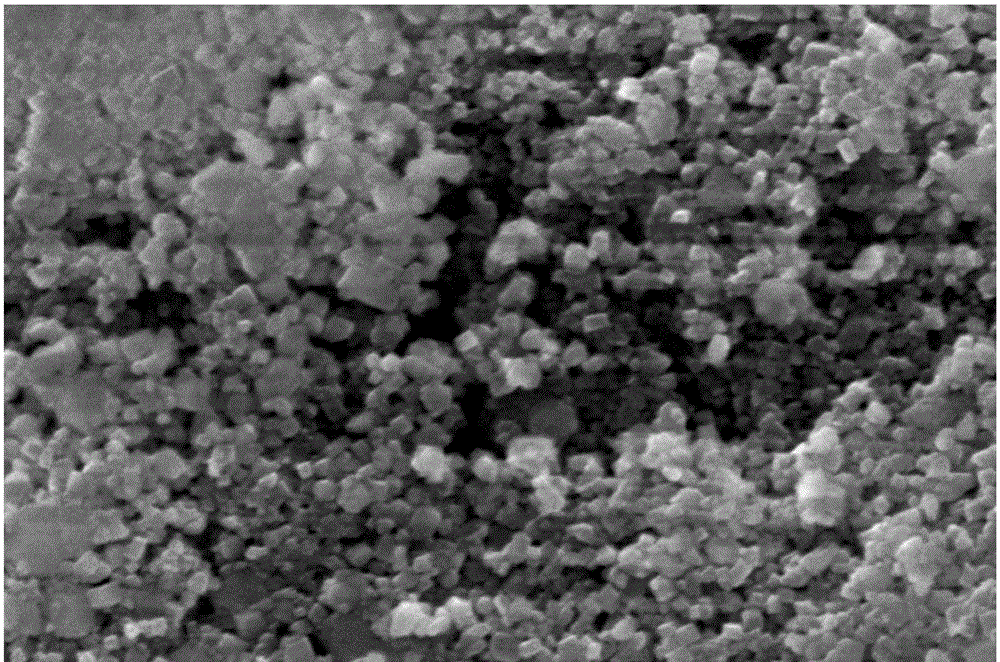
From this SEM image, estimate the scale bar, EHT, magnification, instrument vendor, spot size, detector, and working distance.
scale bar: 3000 nm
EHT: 20 kV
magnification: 50 K X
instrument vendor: FEI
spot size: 3.5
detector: ETD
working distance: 22.7 mm